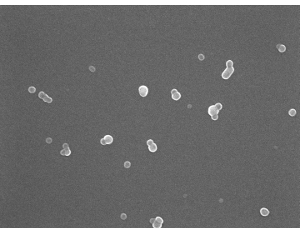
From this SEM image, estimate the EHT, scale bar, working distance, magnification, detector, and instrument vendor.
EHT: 15 kV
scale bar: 100 nm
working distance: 7.7 mm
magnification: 45 K X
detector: SEI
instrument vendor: JEOL